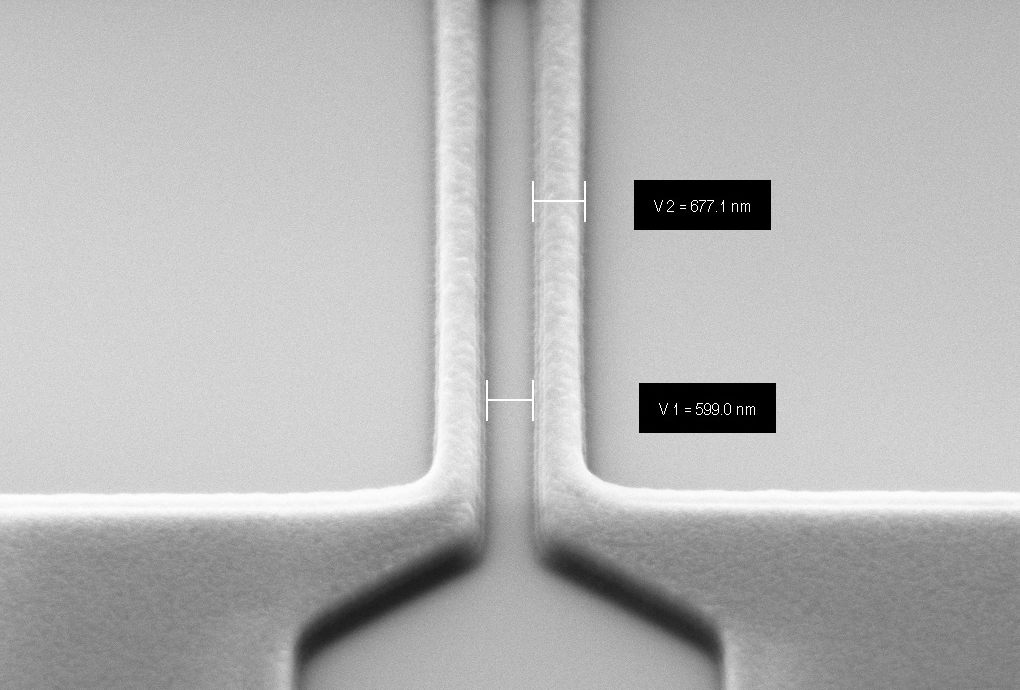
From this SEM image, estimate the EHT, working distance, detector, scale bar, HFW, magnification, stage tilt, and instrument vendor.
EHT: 5 kV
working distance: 5.8 mm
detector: SE2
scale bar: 1000 nm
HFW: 13.33 µm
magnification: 75.01 K X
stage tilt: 36°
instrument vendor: Zeiss